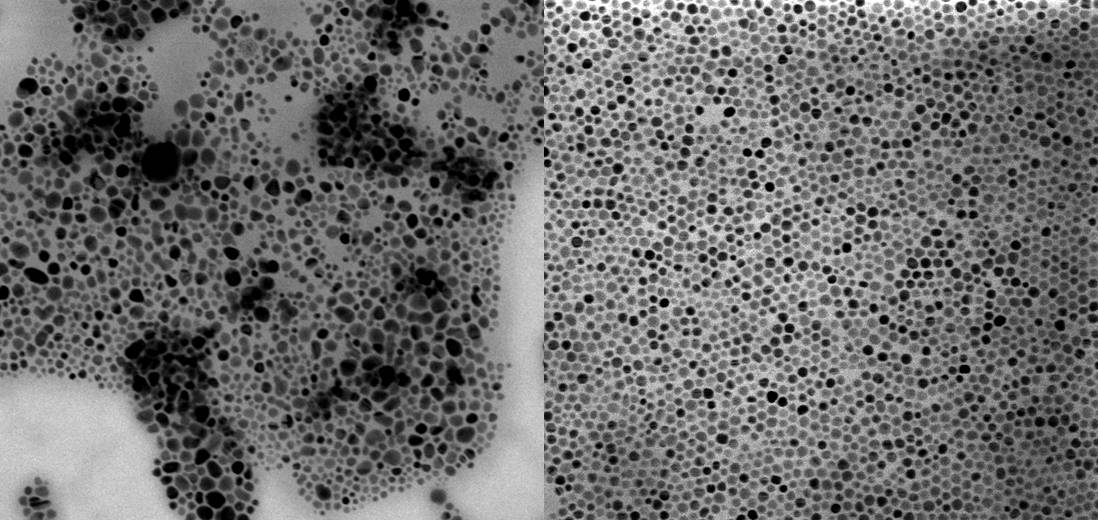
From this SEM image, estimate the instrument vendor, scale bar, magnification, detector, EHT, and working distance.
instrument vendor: JEOL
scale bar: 100 nm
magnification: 100 K X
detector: TED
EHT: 30 kV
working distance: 8.3 mm